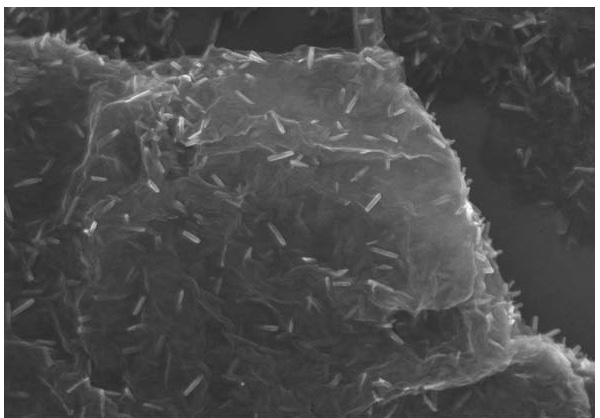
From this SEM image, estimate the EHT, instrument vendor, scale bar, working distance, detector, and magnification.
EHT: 10 kV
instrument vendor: Hitachi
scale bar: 1000 nm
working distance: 6.1 mm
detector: SE(U)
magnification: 50 K X